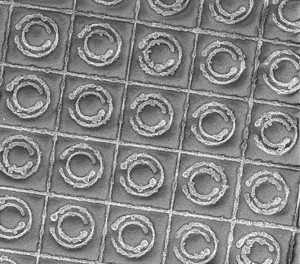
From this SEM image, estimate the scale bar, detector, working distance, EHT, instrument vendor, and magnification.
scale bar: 10000 nm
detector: SEI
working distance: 14 mm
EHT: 5 kV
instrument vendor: JEOL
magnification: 0.95 K X